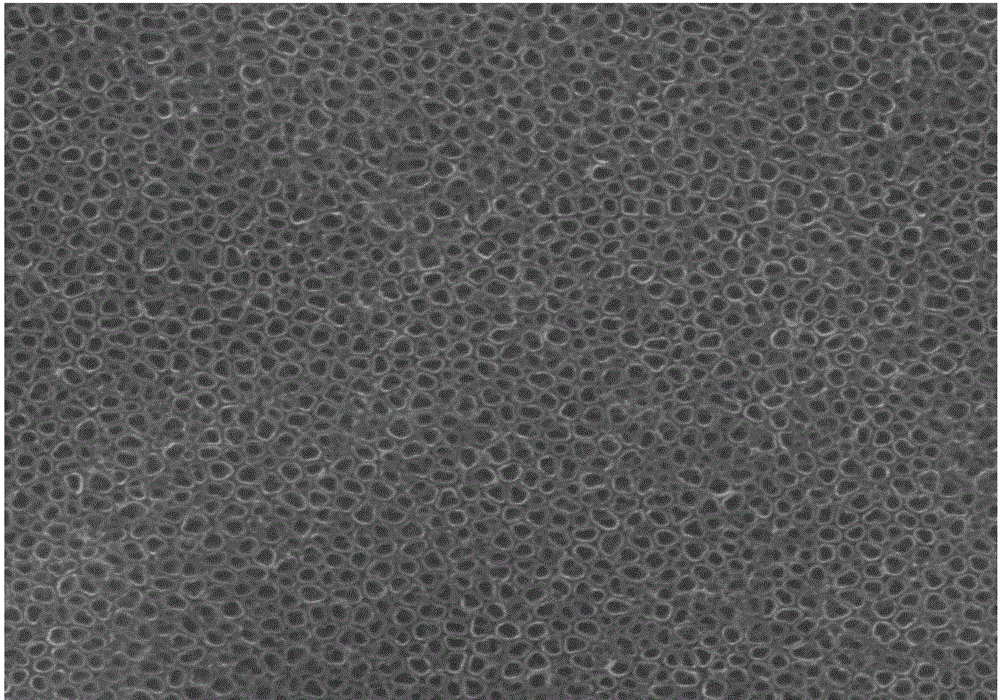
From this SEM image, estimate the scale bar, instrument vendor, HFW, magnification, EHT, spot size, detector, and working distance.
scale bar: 1000 nm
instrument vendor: FEI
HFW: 4.97 µm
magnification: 60 K X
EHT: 30 kV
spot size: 2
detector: ETD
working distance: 10.3 mm